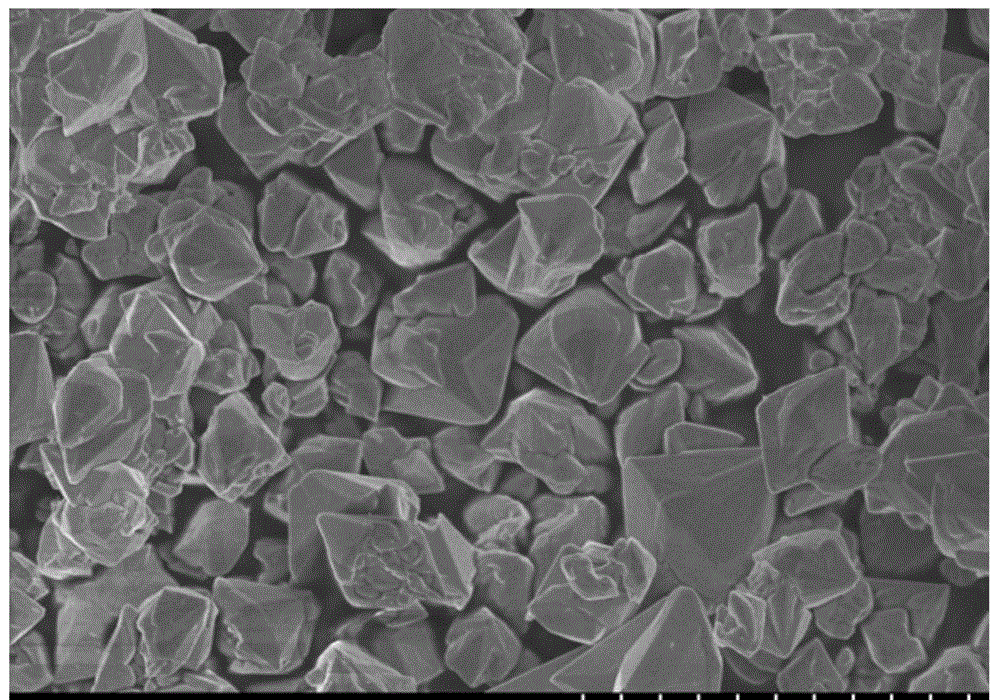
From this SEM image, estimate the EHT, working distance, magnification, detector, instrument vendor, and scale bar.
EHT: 3 kV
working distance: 8.4 mm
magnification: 10 K X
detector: SE(M)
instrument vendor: Hitachi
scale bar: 5000 nm